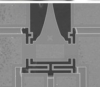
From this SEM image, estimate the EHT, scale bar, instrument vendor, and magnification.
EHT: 15 kV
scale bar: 50000 nm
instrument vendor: JEOL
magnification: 0.3 K X